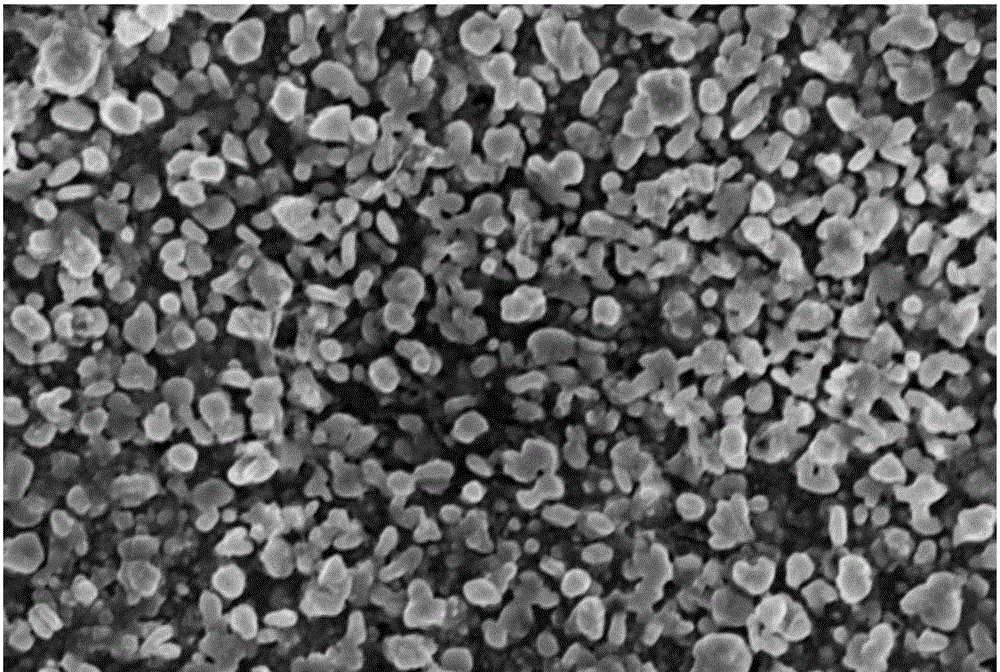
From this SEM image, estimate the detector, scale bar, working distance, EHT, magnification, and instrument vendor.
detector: InLens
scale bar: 1000 nm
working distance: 8.5 mm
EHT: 10 kV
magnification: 8.58 K X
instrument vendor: Zeiss